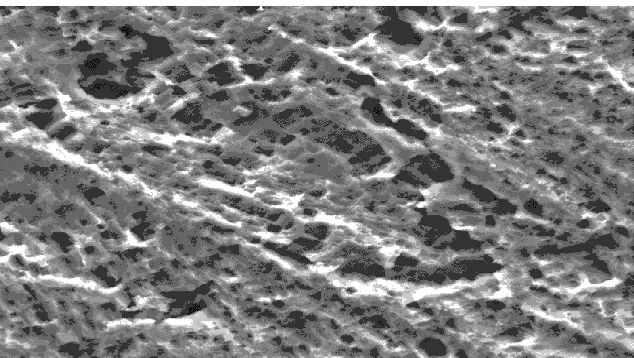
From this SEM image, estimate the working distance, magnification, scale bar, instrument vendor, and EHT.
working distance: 9.4 mm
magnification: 1.555 K X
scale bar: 50000 nm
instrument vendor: FEI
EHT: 25 kV